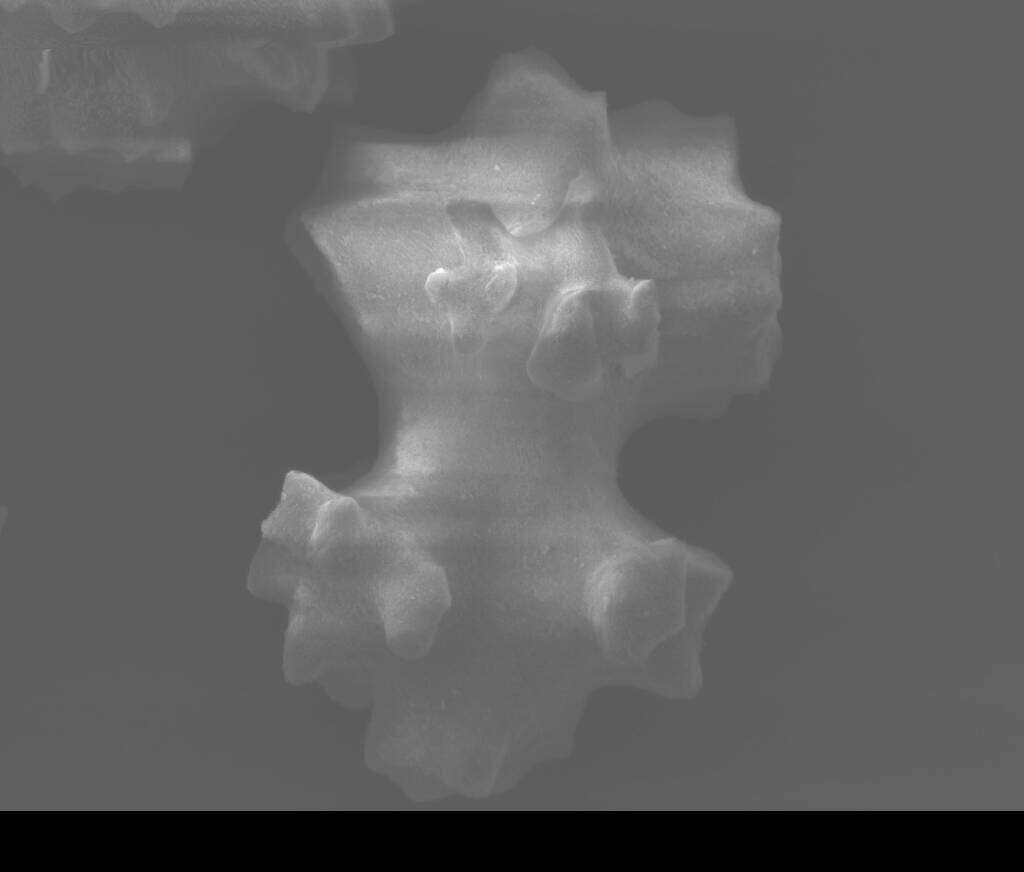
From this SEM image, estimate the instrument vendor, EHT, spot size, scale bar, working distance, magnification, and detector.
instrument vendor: FEI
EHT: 12.5 kV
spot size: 3.5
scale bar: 20000 nm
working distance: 19.9 mm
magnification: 3 K X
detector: SE